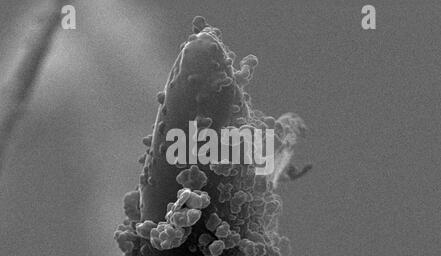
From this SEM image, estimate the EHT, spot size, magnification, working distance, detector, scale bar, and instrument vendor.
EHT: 25 kV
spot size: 3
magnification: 5.169 K X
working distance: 5.1 mm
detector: SE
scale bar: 5000 nm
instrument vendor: FEI